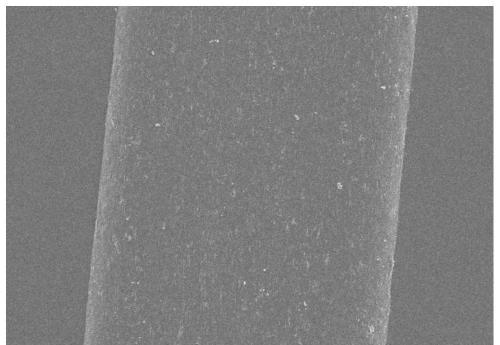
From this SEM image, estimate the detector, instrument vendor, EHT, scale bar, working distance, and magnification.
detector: SE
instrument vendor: Hitachi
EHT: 10 kV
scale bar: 20000 nm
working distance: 8.6 mm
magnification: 2 K X